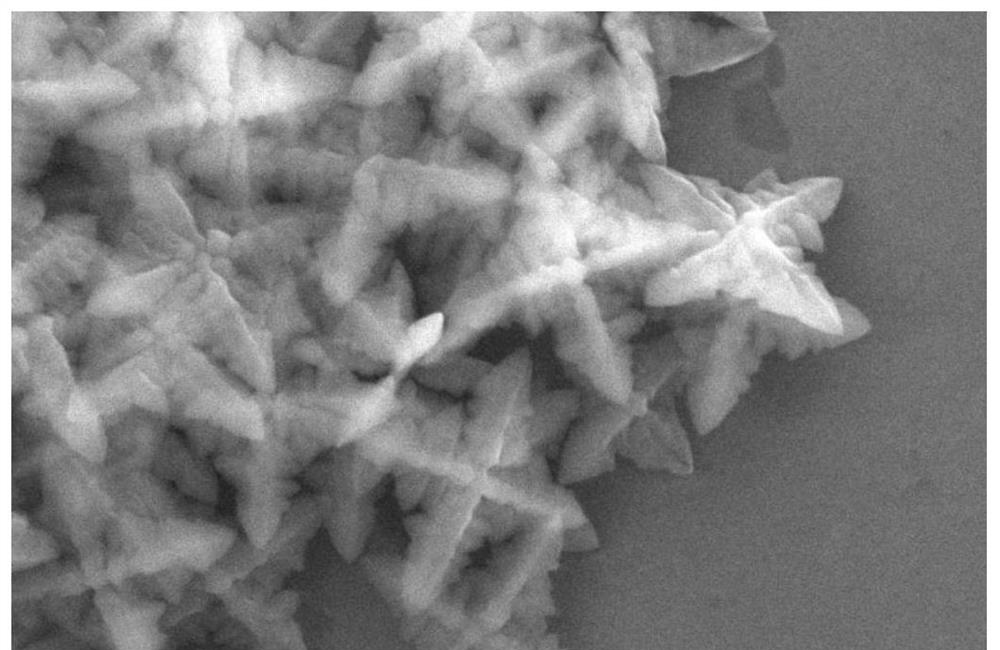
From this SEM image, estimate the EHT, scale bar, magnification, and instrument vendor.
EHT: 20 kV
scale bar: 1000 nm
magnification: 10 K X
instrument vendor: JEOL